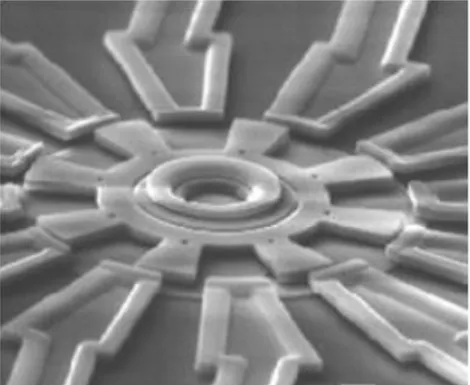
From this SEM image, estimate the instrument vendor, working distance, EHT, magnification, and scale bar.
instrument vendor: JEOL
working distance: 25 mm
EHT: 30 kV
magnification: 0.85 K X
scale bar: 10000 nm